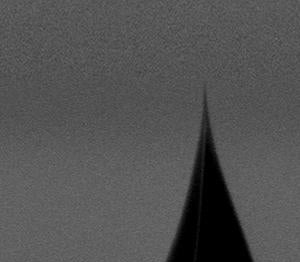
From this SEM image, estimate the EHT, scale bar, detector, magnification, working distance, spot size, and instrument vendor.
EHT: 4 kV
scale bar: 200 nm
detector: TLD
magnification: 80 K X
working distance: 4.7 mm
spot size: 3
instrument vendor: FEI